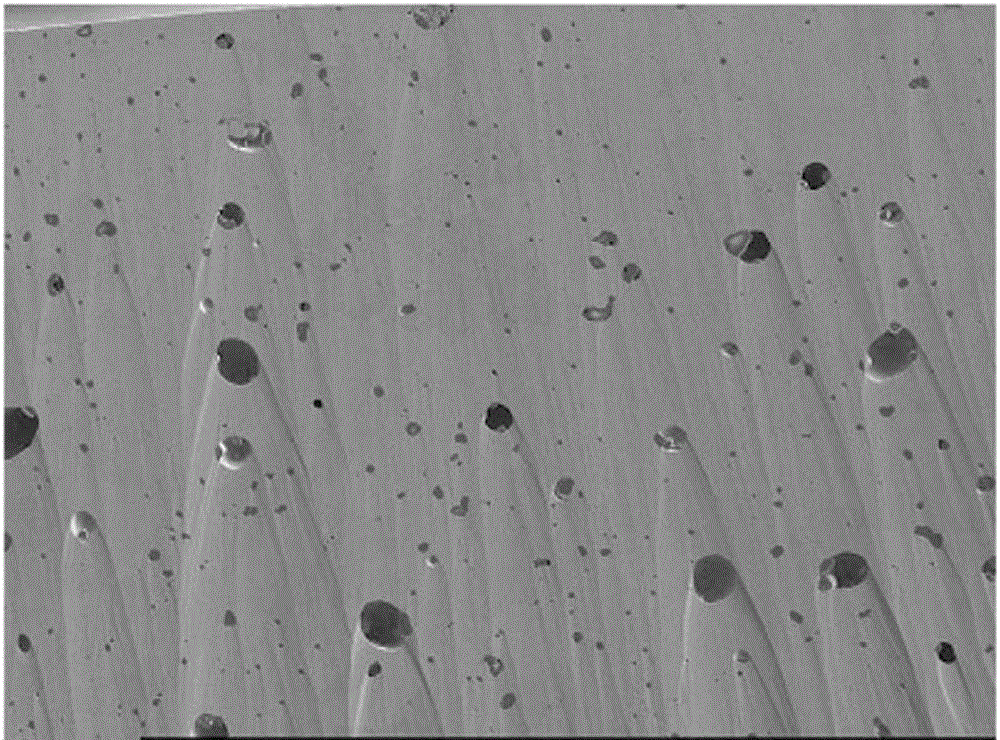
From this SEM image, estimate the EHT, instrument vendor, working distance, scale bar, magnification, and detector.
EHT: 10 kV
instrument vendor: JEOL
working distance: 11 mm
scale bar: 100000 nm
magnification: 0.2 K X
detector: SEI